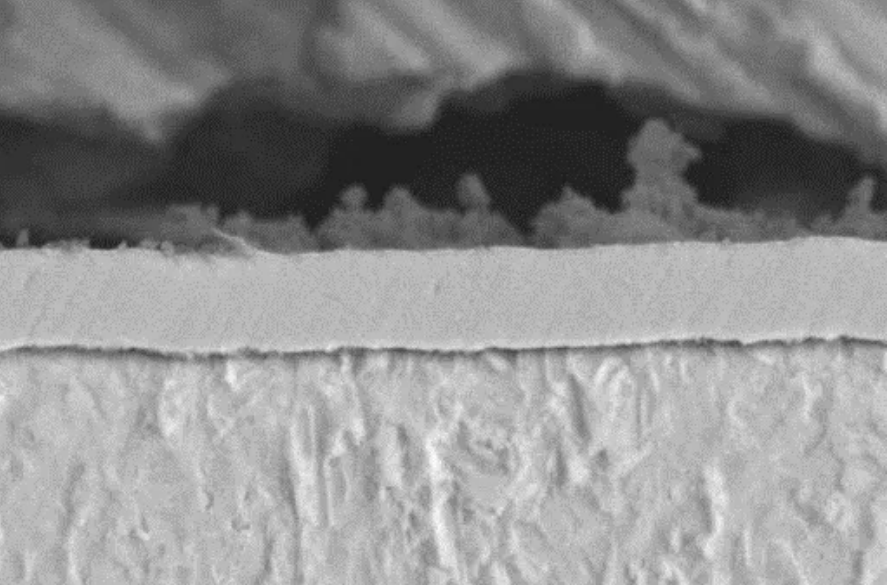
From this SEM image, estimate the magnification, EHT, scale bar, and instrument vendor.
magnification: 3 K X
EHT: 20 kV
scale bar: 5000 nm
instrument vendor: JEOL